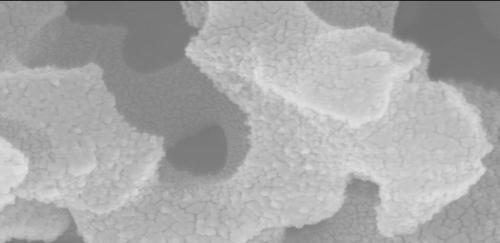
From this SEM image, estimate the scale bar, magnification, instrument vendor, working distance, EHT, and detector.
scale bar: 500 nm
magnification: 100 K X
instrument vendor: Hitachi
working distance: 7.8 mm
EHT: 15 kV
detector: SE(M)